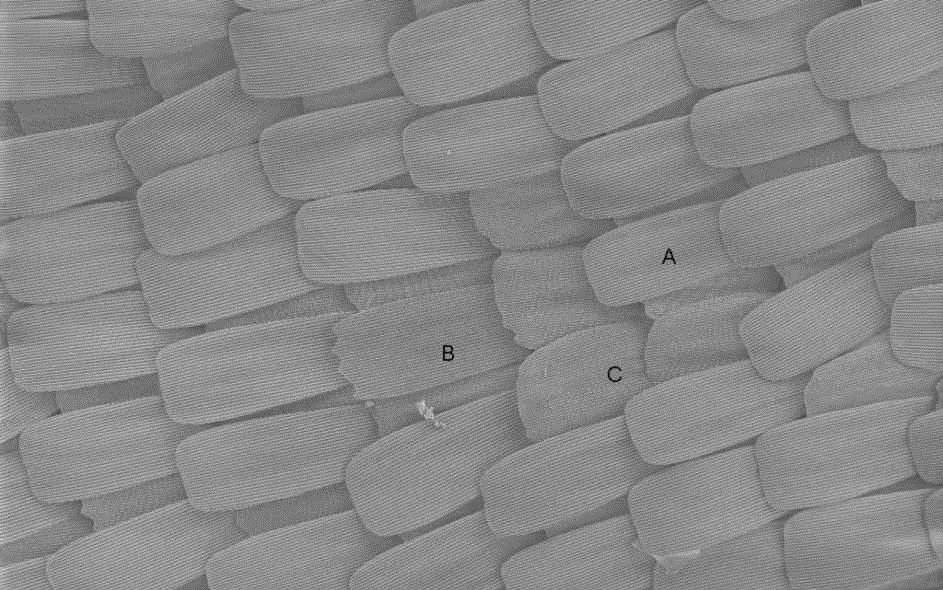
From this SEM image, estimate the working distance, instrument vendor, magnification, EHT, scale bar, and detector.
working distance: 3 mm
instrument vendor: Zeiss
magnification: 0.5 K X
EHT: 1 kV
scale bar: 20000 nm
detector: InLens